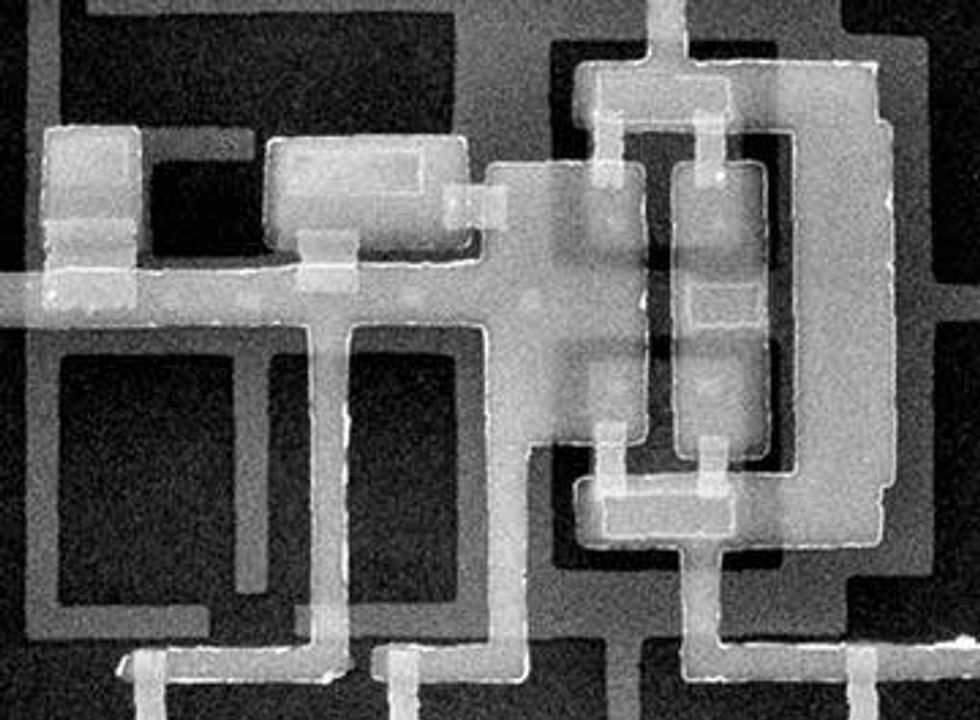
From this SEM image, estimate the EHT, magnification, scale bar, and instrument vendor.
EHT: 30 kV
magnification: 3 K X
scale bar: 10000 nm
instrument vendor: JEOL